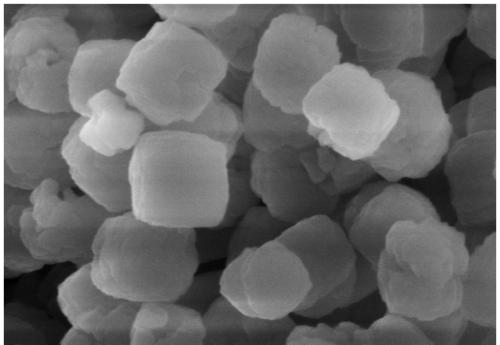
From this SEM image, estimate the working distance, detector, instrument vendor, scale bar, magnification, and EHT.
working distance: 11.1 mm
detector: SE(M)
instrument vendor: Hitachi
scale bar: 1000 nm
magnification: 50 K X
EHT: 5 kV